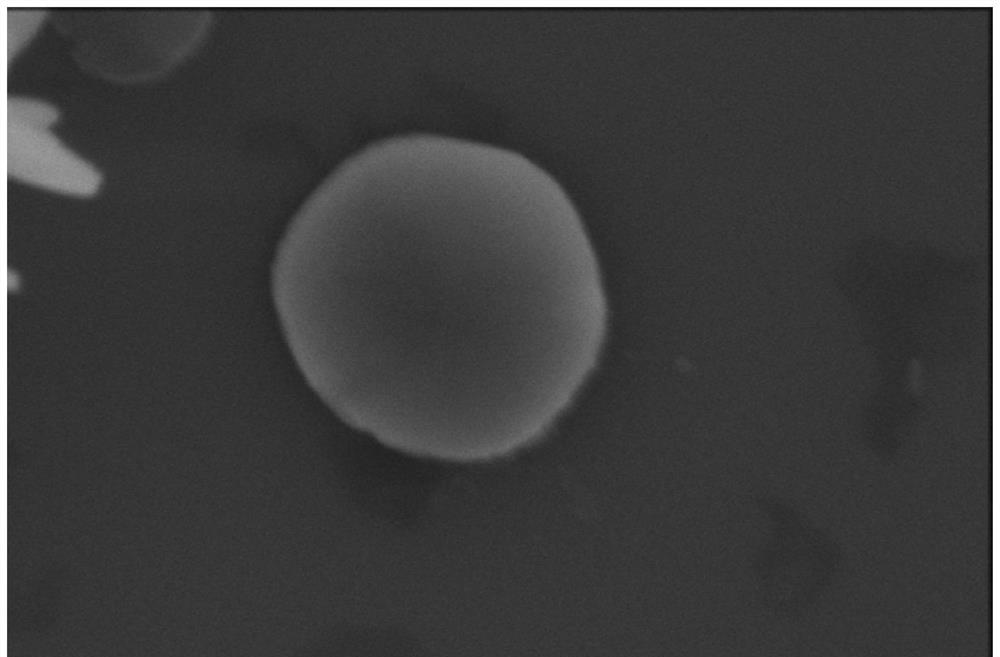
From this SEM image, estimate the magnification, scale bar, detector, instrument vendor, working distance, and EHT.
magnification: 250 K X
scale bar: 500 nm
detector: TLD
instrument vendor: FEI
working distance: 4 mm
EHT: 5 kV